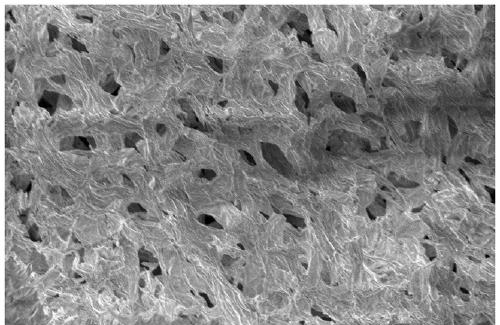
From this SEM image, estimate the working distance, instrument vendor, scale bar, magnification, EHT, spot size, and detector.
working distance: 11.5 mm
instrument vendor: FEI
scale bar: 400000 nm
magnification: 0.4 K X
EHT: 10 kV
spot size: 3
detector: ETD